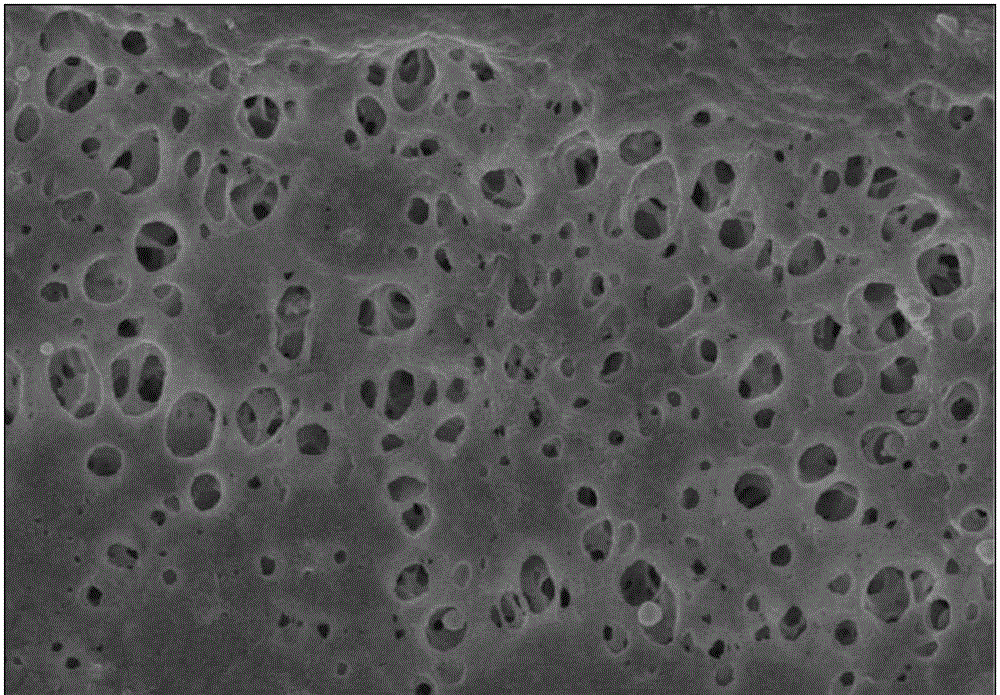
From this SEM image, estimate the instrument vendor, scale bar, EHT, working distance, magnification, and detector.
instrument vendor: Hitachi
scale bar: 5000 nm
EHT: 4 kV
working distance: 8.7 mm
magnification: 10 K X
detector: SE(U)